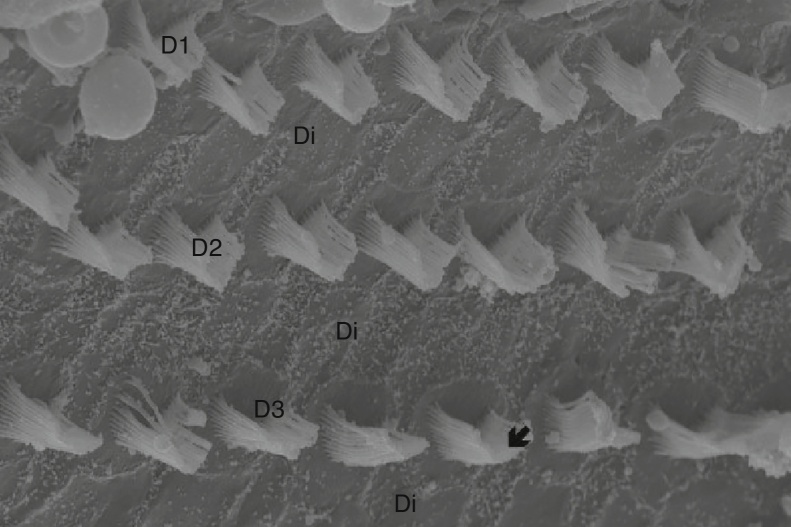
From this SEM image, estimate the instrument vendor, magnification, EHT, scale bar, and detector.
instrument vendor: Zeiss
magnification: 10 K X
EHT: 20 kV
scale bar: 2000 nm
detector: SE1